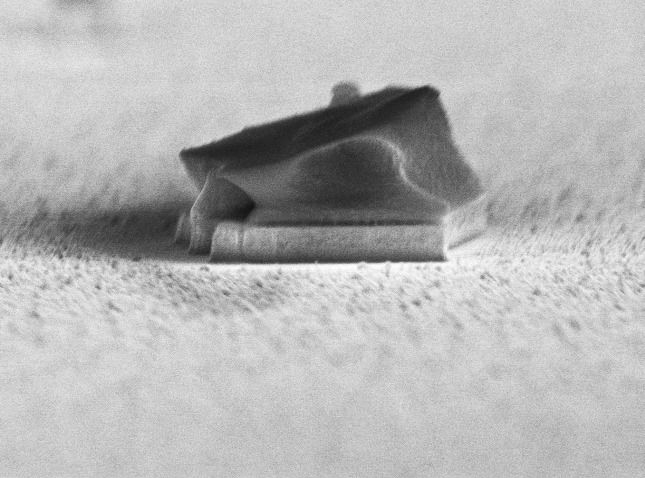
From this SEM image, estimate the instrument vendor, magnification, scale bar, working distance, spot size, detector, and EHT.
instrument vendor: FEI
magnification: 14.522 K X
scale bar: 2000 nm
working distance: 6.2 mm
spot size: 2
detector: TLD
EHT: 10 kV